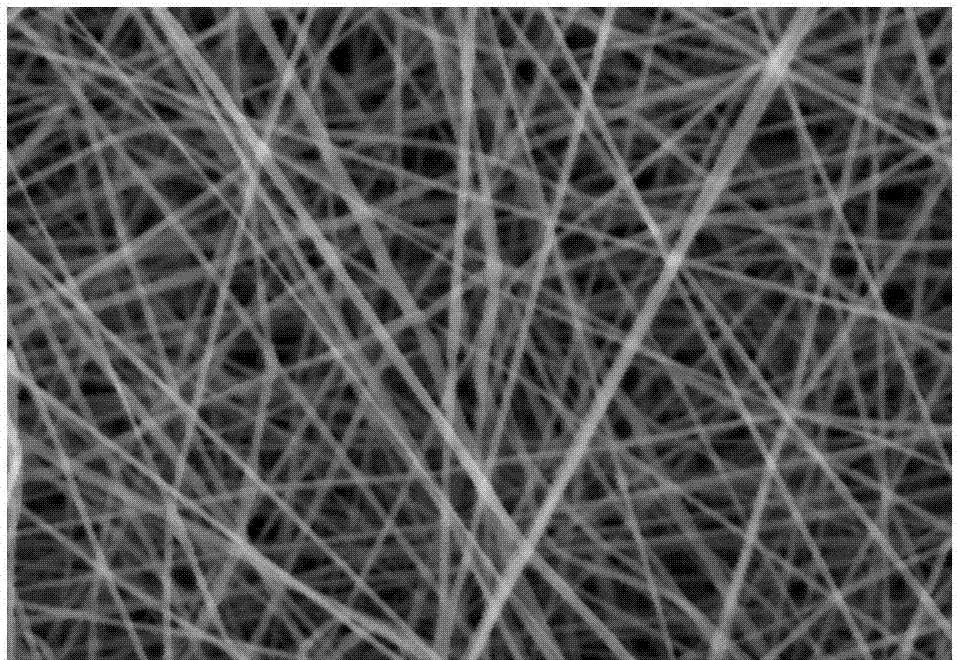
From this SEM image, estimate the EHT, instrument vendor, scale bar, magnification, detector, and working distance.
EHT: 15 kV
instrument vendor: Hitachi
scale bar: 10000 nm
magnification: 5 K X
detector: SE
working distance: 13.7 mm